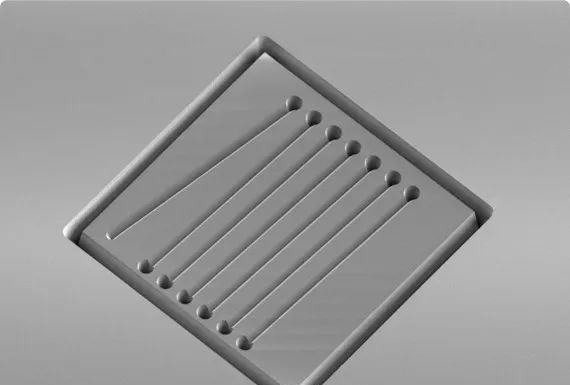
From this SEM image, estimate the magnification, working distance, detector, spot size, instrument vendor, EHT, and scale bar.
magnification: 0.095 K X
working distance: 35 mm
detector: SEI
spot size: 49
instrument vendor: JEOL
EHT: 5 kV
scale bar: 200000 nm